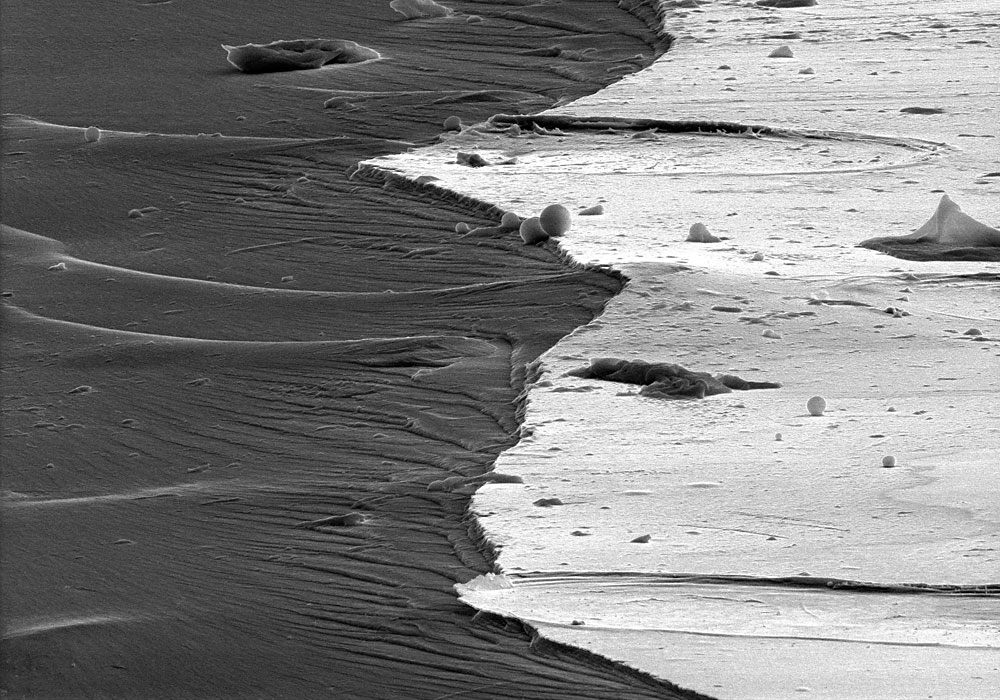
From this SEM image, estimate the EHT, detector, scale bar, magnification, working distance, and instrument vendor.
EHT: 15 kV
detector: SE(M)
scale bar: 50000 nm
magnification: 0.9 K X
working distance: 13.4 mm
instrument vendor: Hitachi